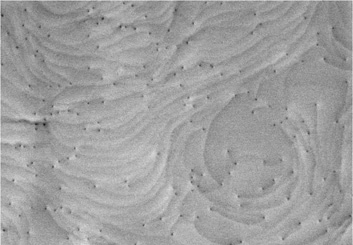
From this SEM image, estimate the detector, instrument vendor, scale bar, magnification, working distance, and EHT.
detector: BSE-ALL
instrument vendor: Hitachi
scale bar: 2000 nm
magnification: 25 K X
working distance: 5.6 mm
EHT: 7 kV